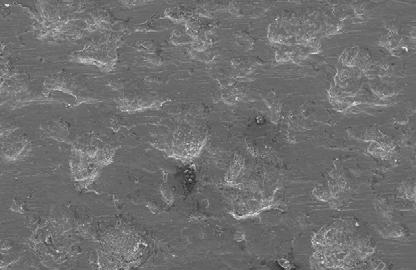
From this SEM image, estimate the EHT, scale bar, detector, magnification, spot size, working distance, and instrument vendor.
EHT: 20 kV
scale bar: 200000 nm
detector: SE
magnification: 0.2 K X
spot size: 4.4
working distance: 10.9 mm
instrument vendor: FEI